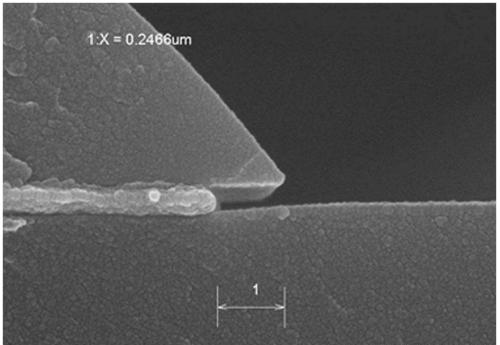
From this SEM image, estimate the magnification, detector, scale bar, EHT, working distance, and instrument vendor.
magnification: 70 K X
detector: SE(U)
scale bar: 500 nm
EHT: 15 kV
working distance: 8.7 mm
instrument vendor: Hitachi